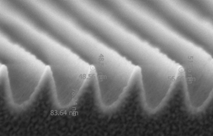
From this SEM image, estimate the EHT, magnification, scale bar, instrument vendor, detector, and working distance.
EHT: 2 kV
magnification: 100 K X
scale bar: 1000 nm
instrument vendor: FEI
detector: T1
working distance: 9.4 mm